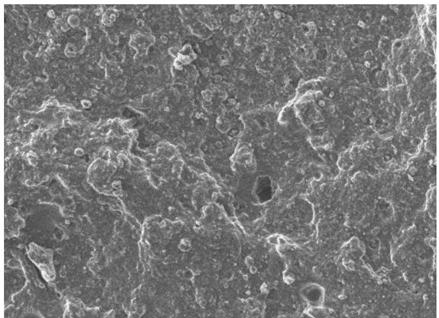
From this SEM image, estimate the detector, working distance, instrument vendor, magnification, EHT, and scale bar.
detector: LEI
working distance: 8 mm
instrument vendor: JEOL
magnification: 0.5 K X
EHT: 8 kV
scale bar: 10000 nm